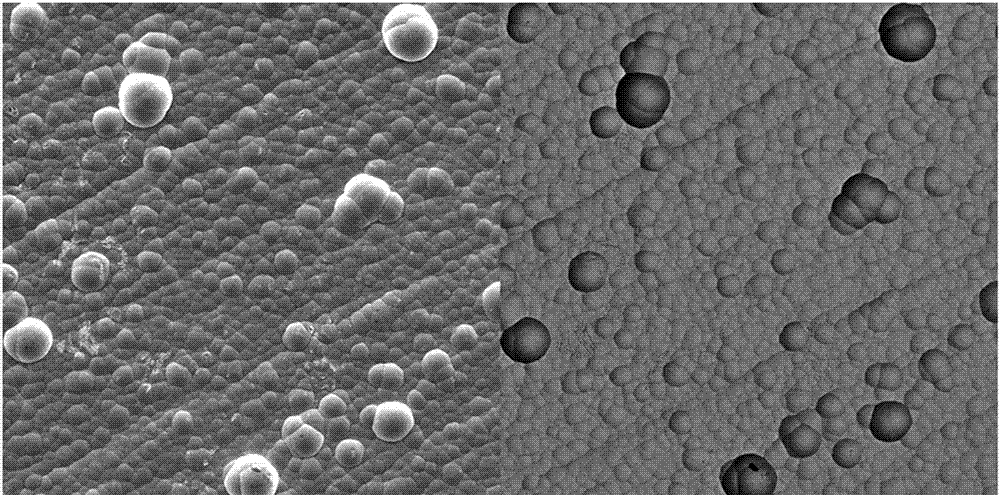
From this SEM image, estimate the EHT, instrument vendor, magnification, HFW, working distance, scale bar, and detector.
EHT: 20 kV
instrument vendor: Tescan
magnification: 0.5 K X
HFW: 382 µm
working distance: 12.97 mm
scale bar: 200000 nm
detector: SE, BSE